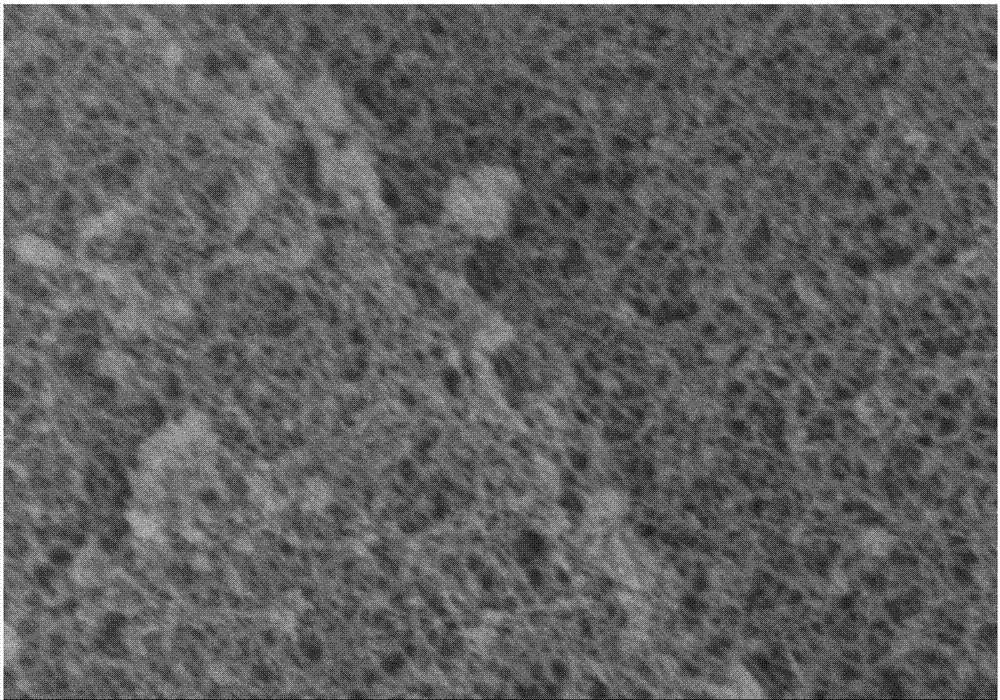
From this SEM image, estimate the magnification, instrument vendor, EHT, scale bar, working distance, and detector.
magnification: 50 K X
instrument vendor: Hitachi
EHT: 5 kV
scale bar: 1000 nm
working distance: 7.8 mm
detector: SE(M)